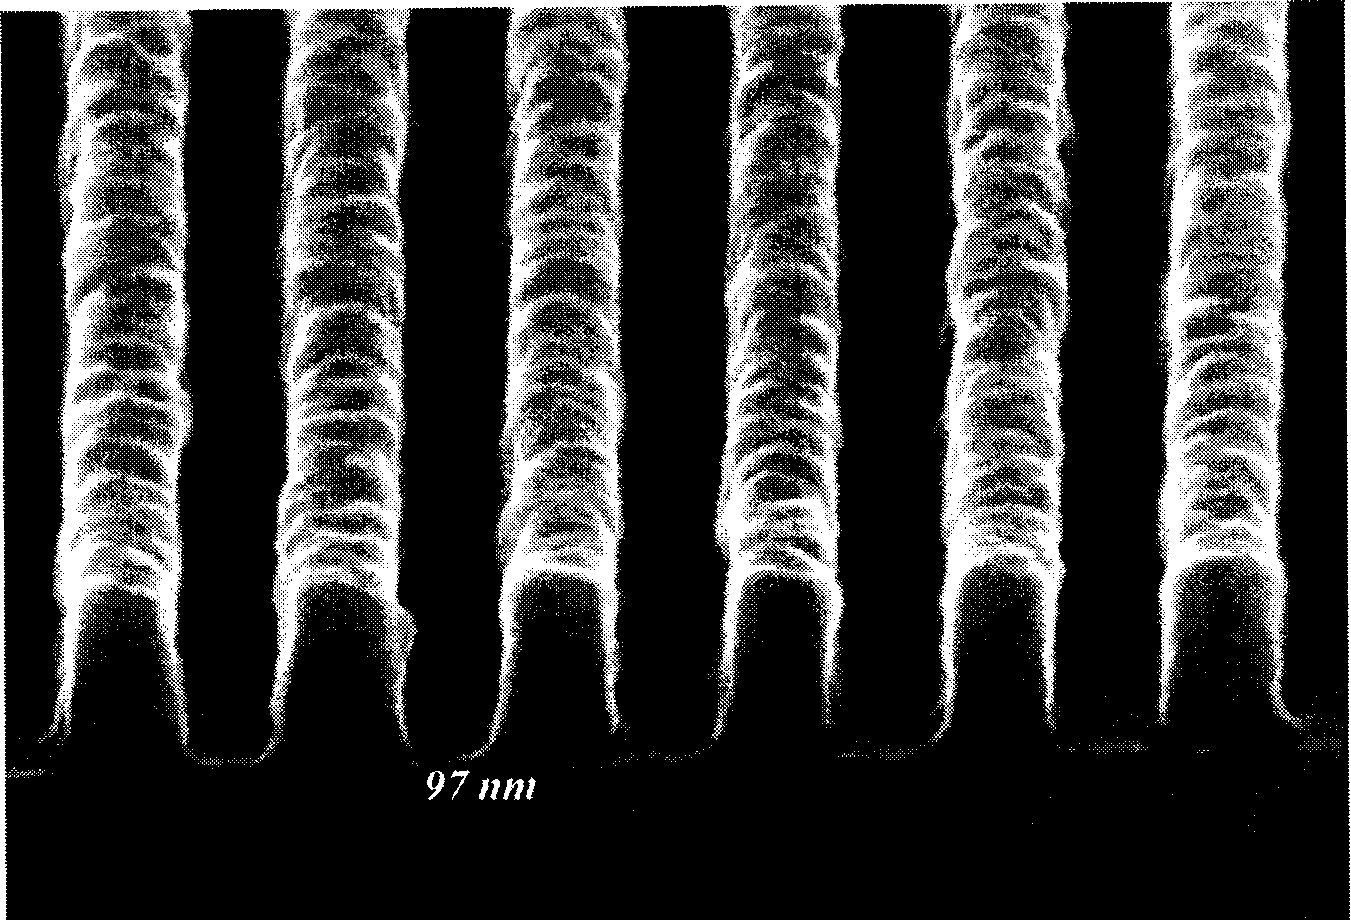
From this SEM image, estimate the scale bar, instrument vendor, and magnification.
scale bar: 272 nm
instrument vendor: Hitachi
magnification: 110 K X